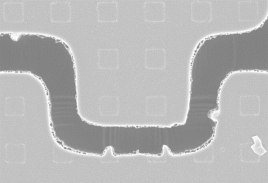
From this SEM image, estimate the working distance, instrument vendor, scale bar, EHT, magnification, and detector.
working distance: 3.8 mm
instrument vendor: Zeiss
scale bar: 1000 nm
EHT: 5 kV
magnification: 5 K X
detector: InLens